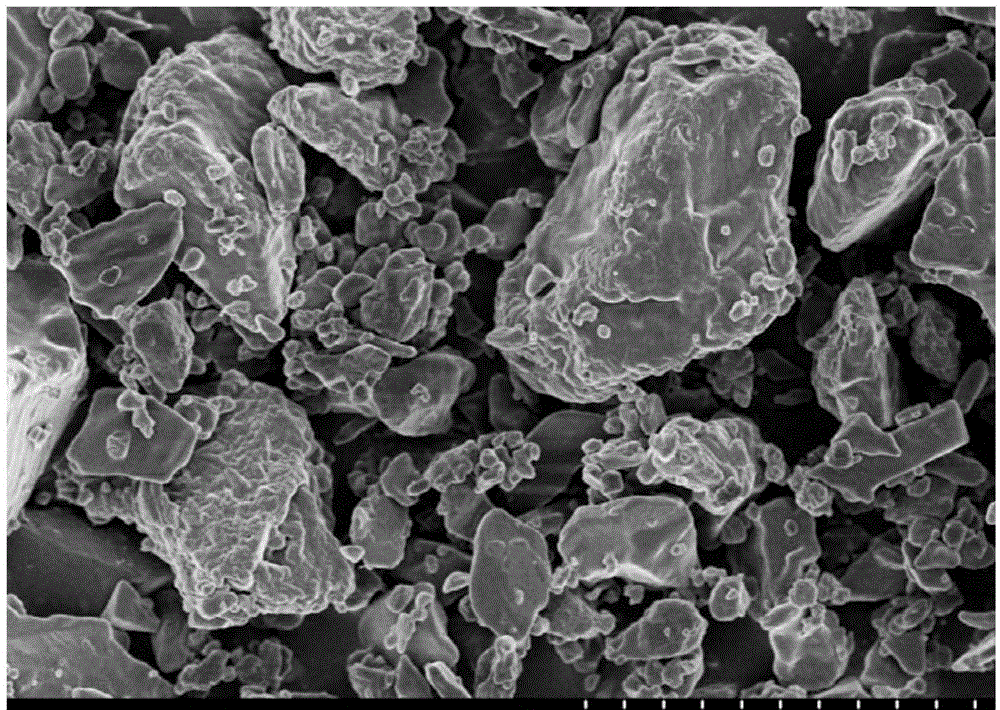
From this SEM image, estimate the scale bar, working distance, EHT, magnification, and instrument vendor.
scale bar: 10000 nm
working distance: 2.5 mm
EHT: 3 kV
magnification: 5 K X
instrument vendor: Hitachi